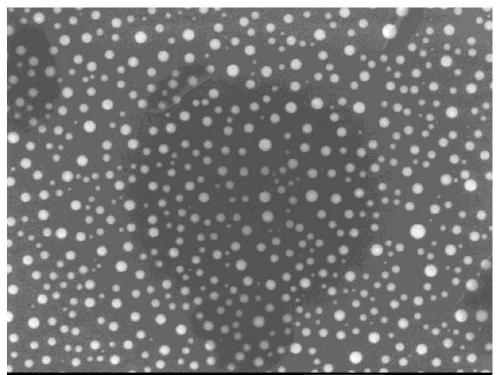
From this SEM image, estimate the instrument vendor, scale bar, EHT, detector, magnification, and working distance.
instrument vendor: JEOL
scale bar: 1000 nm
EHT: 10 kV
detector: SEI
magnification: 20 K X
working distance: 8 mm